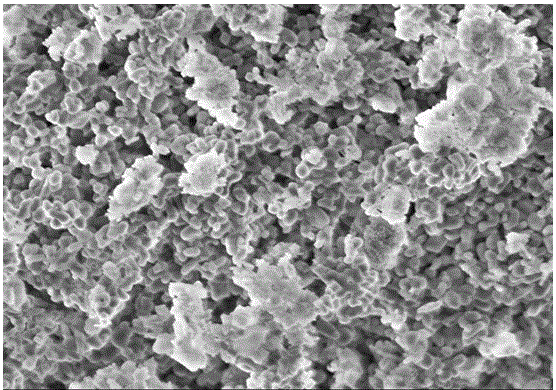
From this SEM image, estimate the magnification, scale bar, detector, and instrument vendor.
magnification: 10 K X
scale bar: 5000 nm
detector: SE(U)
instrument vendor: Hitachi